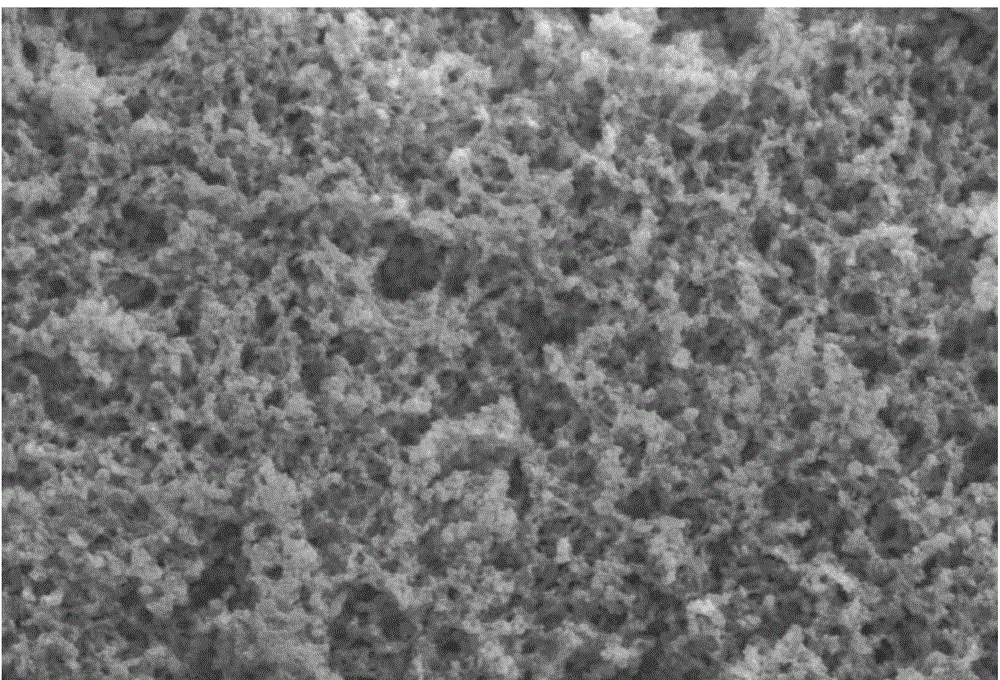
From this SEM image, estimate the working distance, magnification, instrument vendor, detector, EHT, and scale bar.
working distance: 10.1 mm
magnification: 2 K X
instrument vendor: Hitachi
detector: SE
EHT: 15 kV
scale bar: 20000 nm